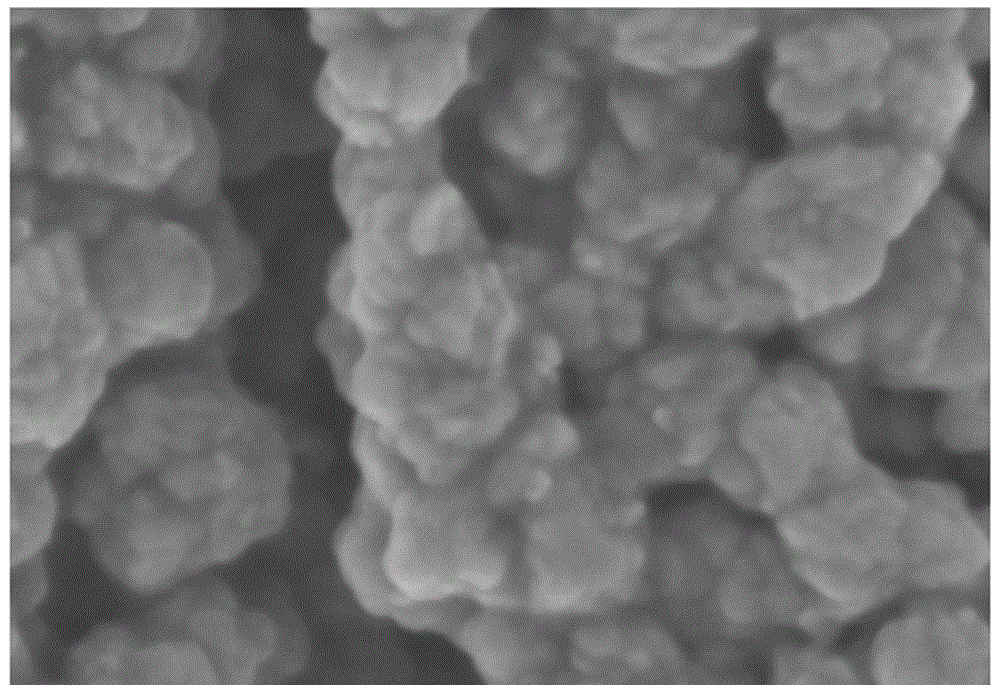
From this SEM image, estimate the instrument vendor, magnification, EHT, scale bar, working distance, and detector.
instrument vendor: Hitachi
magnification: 200 K X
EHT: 3 kV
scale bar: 200 nm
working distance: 4.2 mm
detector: SE(M)